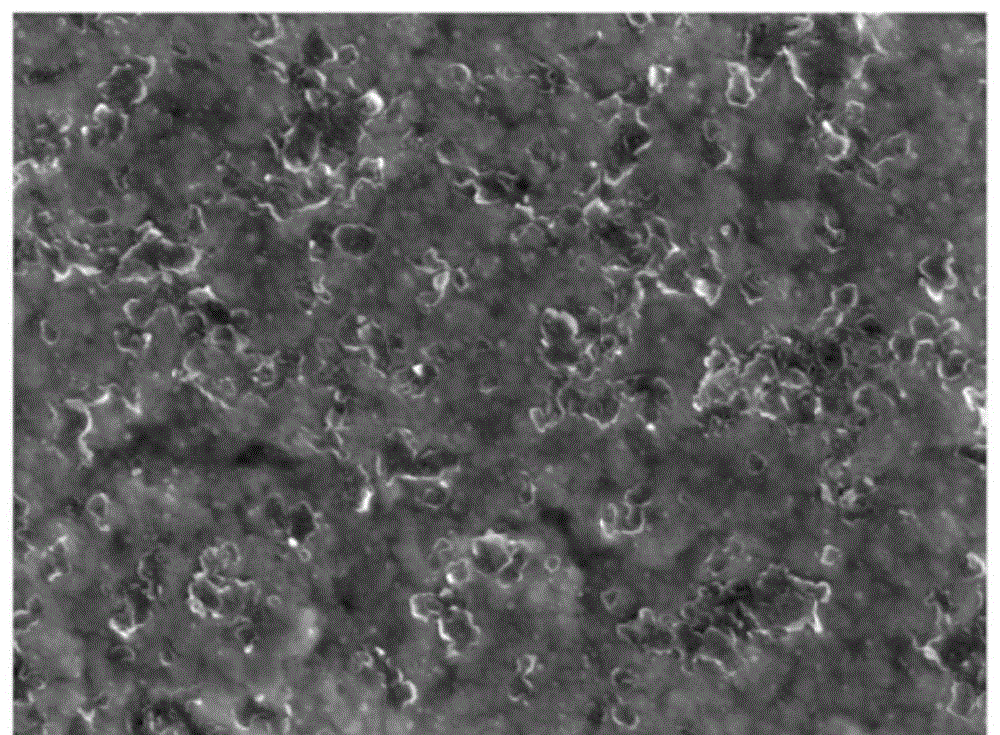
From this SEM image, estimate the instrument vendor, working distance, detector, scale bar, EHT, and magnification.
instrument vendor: JEOL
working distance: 9 mm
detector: SEI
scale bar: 1000 nm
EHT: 10 kV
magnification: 20 K X